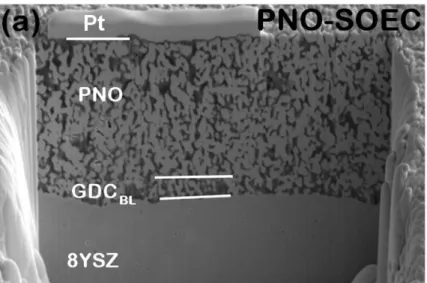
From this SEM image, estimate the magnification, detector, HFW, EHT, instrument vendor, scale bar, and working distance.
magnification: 2.5 K X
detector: ETD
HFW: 50.8 µm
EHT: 5 kV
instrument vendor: FEI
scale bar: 10000 nm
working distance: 4 mm